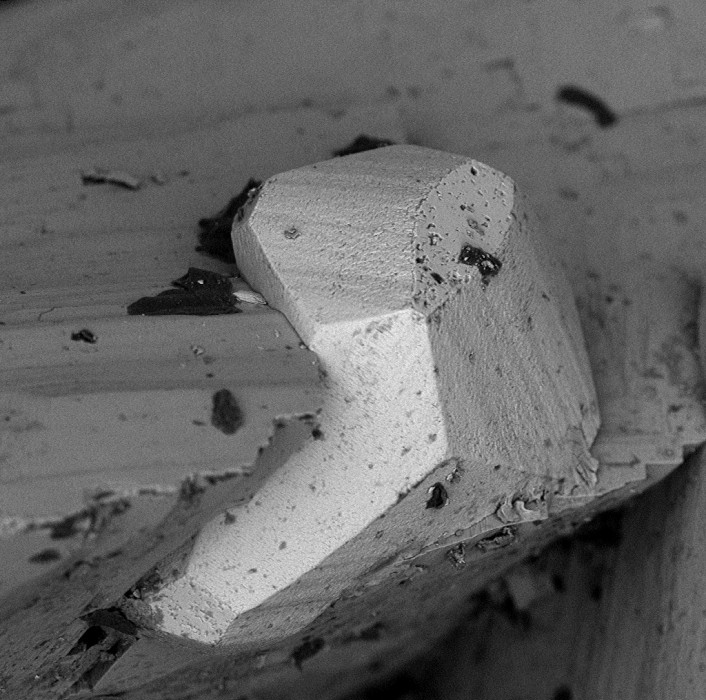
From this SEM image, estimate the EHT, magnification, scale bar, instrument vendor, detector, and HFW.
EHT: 10 kV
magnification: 1 K X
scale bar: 80000 nm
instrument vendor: other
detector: BSD Full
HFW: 271 µm